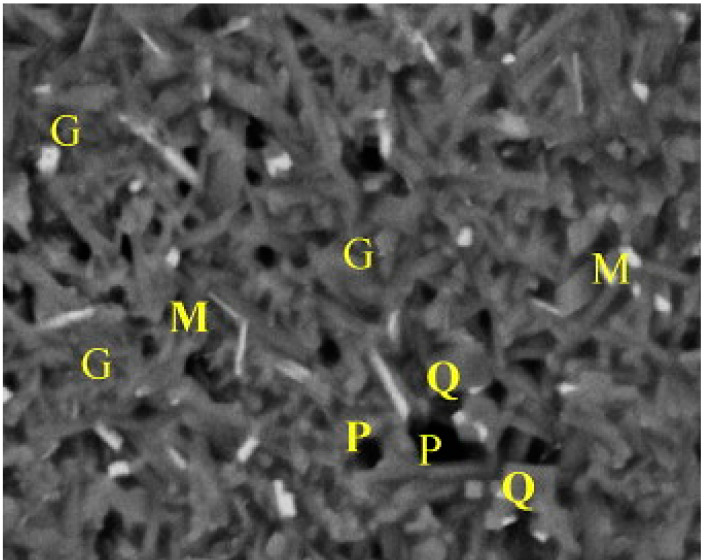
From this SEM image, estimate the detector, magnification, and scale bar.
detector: BSE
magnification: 3 K X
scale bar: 8000 nm